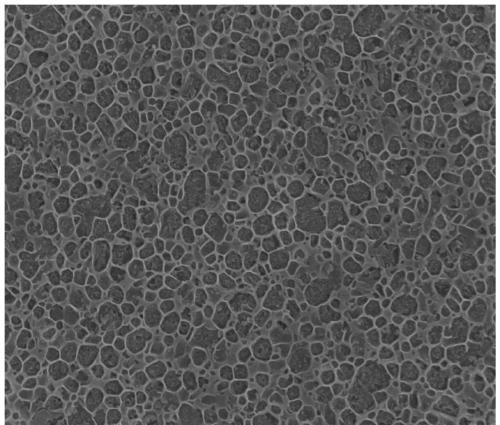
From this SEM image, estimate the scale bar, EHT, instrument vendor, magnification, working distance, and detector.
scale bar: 2000 nm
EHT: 10 kV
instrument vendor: FEI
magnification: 40 K X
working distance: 6.4 mm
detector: TLD